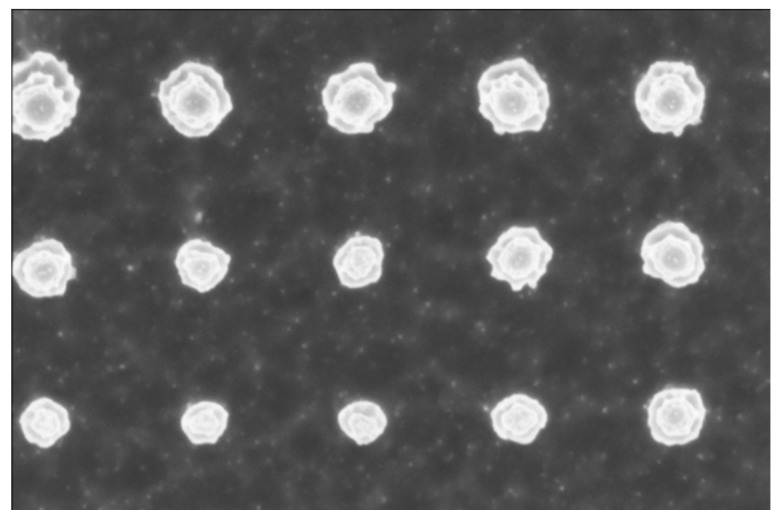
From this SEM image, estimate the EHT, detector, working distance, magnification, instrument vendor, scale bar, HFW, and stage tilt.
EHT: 2 kV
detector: TLD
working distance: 4.1 mm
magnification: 65 K X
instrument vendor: FEI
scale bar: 500 nm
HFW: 1.95 µm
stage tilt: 0°